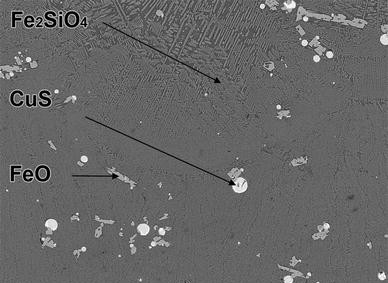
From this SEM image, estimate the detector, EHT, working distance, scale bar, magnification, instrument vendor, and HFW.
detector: BSE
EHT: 20 kV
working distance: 15.04 mm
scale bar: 100000 nm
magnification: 0.5 K X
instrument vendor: Tescan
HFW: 555 µm